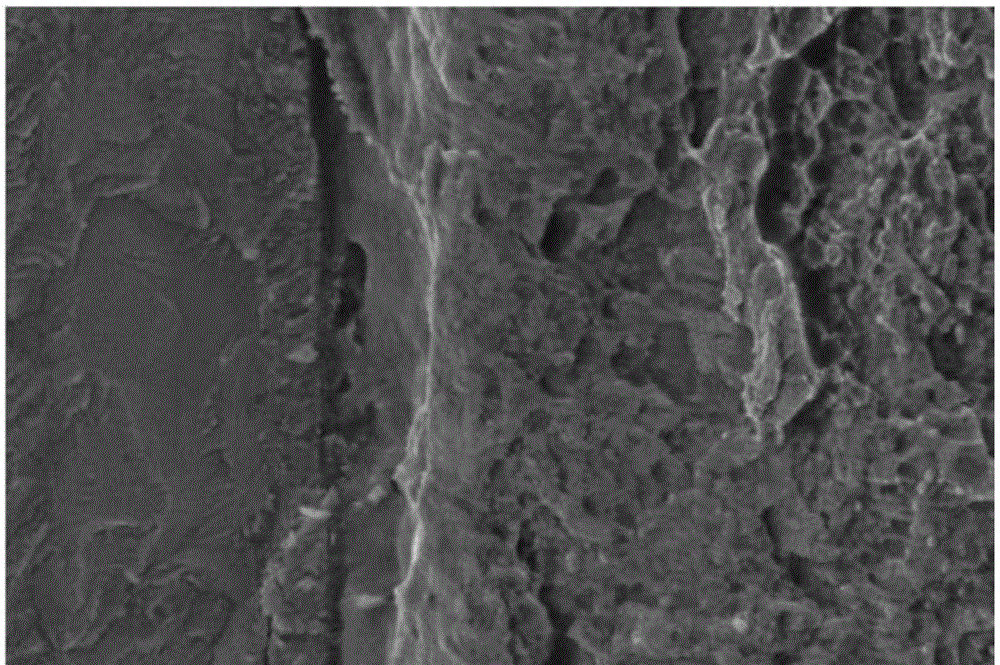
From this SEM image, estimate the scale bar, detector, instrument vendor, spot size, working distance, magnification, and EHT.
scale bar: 5000 nm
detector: SE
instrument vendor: FEI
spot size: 3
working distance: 6.2 mm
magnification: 5 K X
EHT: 12 kV